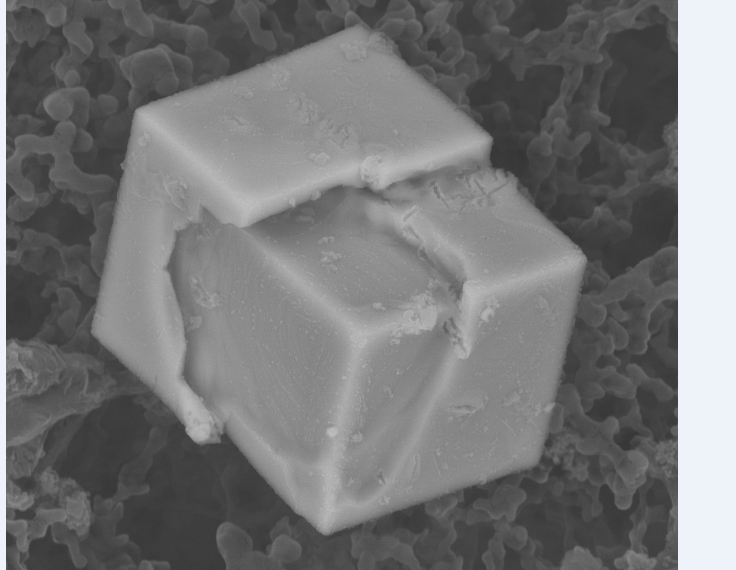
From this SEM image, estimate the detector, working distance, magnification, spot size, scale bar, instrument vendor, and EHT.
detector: BSED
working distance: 10 mm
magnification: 25 K X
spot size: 4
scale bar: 3000 nm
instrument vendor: FEI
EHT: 15 kV